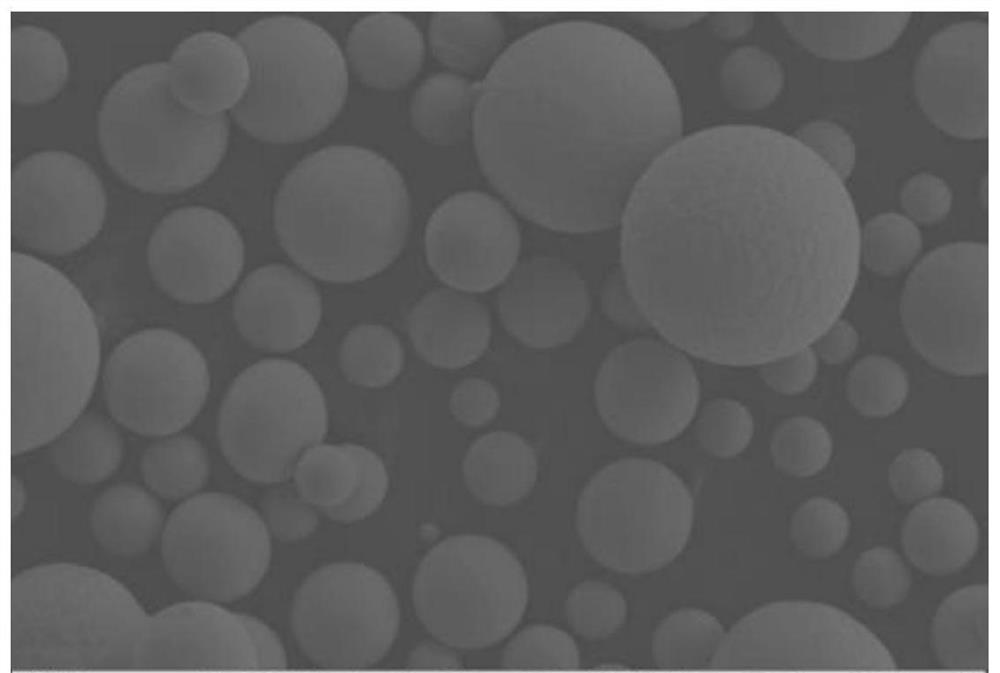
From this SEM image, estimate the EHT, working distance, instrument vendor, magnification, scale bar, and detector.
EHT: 20 kV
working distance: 7.8 mm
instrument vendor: Zeiss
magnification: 0.2 K X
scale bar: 20000 nm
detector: SE2